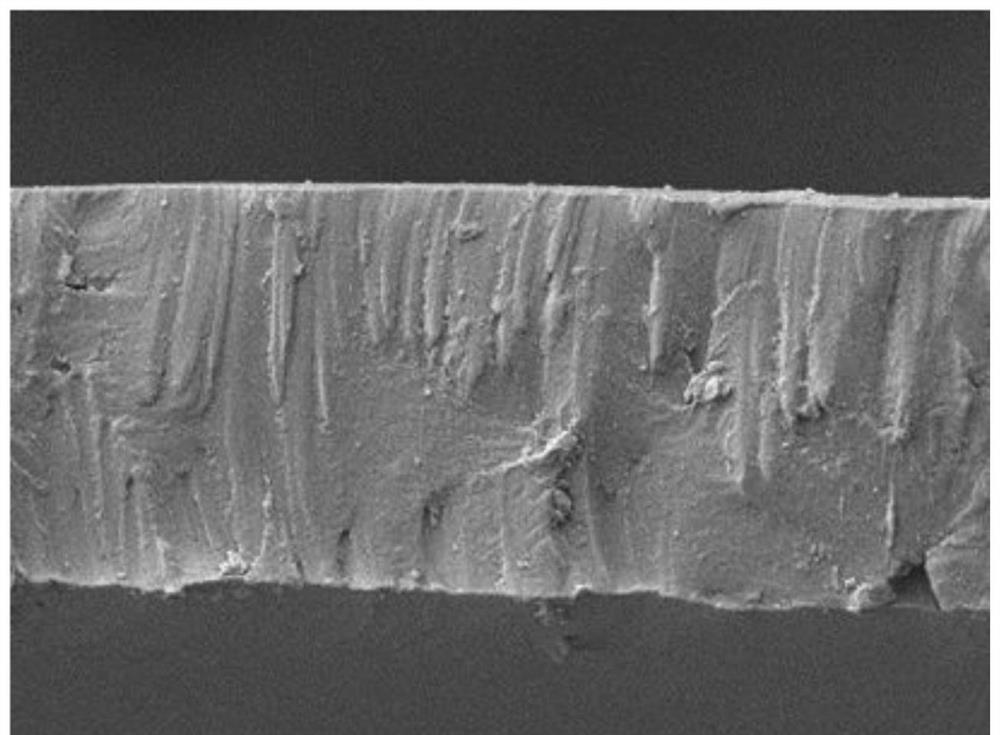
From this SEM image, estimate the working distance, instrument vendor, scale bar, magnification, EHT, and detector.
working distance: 11.5 mm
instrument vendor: JEOL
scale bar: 10000 nm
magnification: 2 K X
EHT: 5 kV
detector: SEM